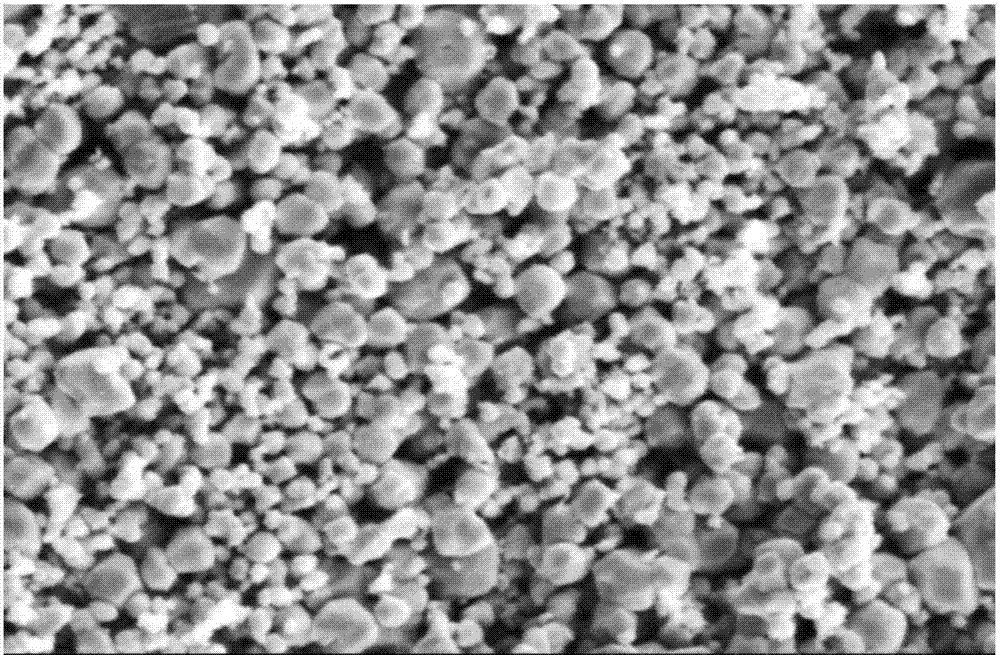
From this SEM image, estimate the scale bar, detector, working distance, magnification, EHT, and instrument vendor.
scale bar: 10000 nm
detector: SE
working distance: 11.5 mm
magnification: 4 K X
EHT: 20 kV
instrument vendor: Hitachi